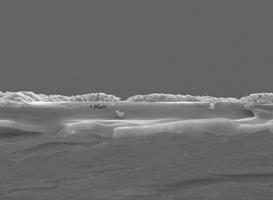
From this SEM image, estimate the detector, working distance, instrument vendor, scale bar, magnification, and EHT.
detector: SEI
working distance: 10.1 mm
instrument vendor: JEOL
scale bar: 10000 nm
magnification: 2 K X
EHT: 15 kV